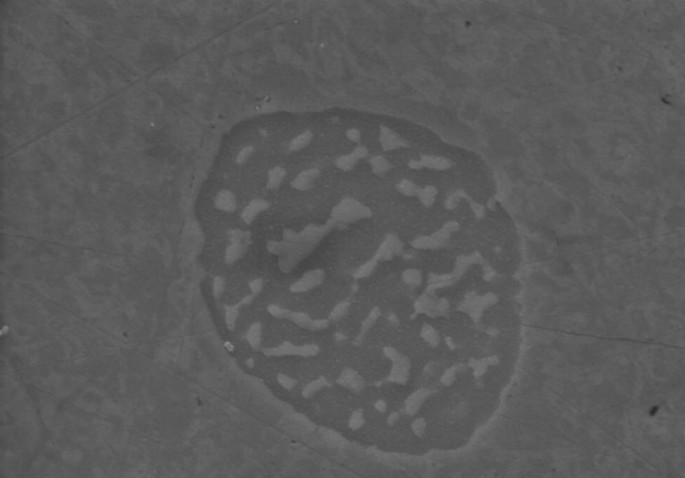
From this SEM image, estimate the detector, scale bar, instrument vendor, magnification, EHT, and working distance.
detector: SE(M)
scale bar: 5000 nm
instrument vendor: Hitachi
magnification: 10 K X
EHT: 15 kV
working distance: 15.2 mm